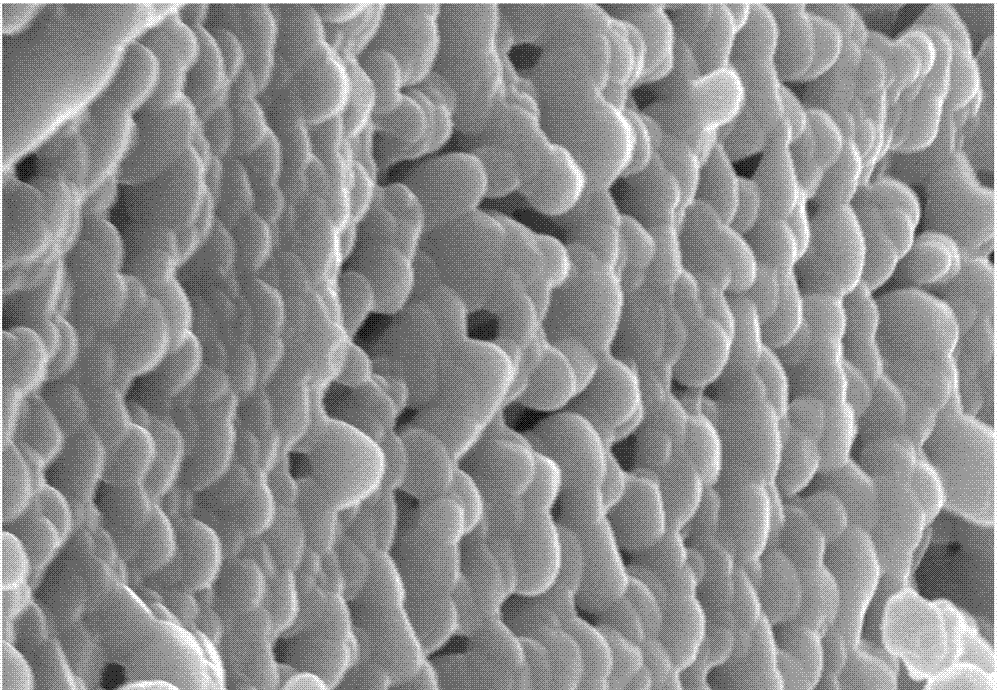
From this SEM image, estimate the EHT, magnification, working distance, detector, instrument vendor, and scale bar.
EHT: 20 kV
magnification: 20 K X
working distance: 15.1 mm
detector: SE(U)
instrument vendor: Hitachi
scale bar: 2000 nm